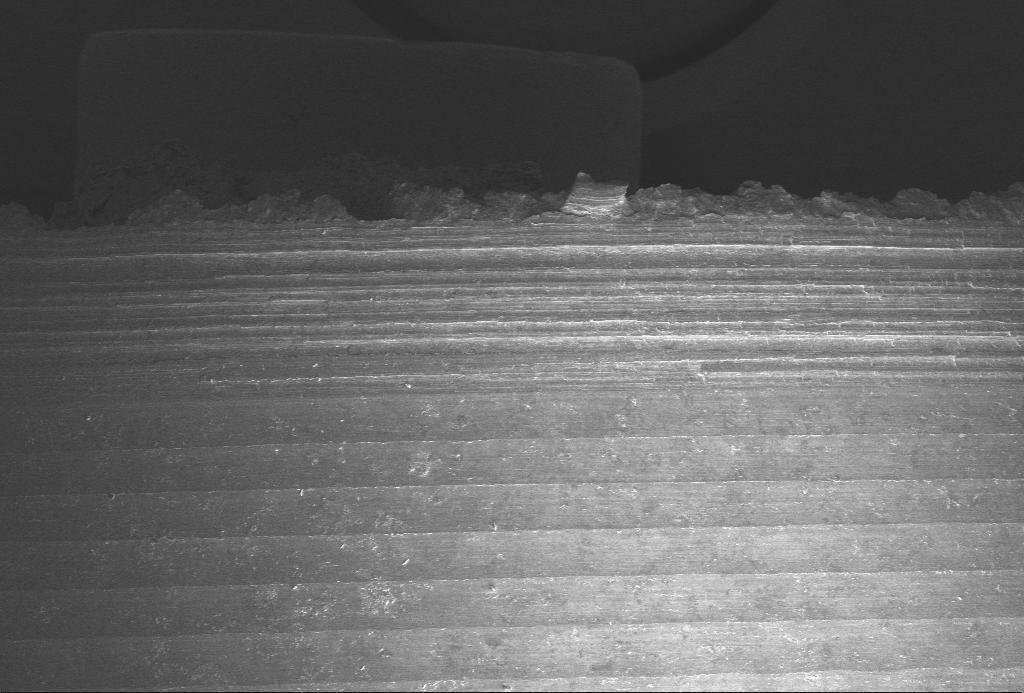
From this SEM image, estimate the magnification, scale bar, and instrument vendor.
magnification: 0.05 K X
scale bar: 200000 nm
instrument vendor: Zeiss